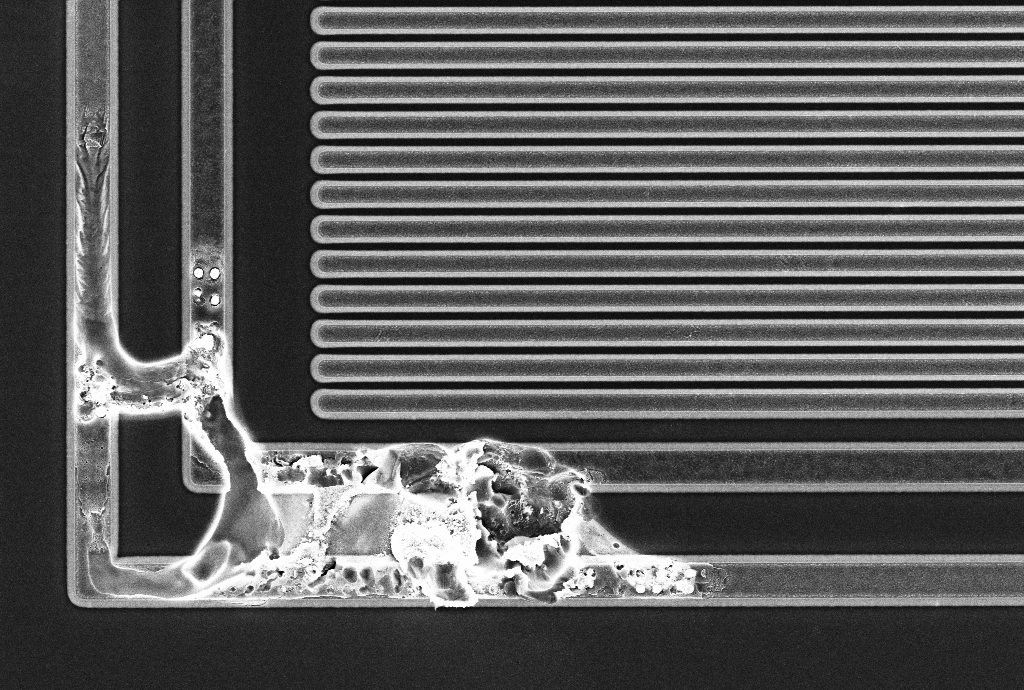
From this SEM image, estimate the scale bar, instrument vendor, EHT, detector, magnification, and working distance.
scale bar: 2000 nm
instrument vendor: Zeiss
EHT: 5 kV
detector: InLens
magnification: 3.05 K X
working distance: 5.1 mm